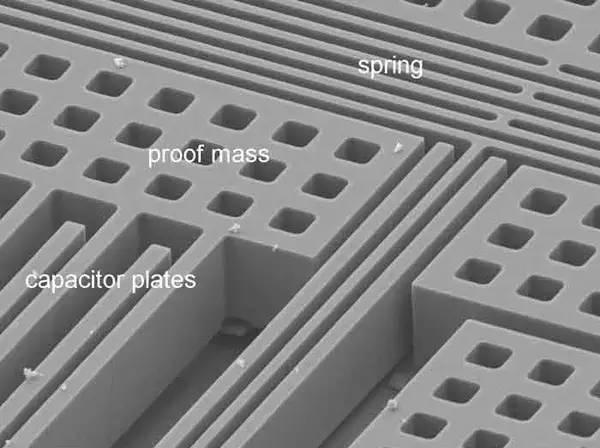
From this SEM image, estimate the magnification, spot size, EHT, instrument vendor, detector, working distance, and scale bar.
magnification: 1.5 K X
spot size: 3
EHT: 10 kV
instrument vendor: FEI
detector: SE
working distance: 14.6 mm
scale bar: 20000 nm